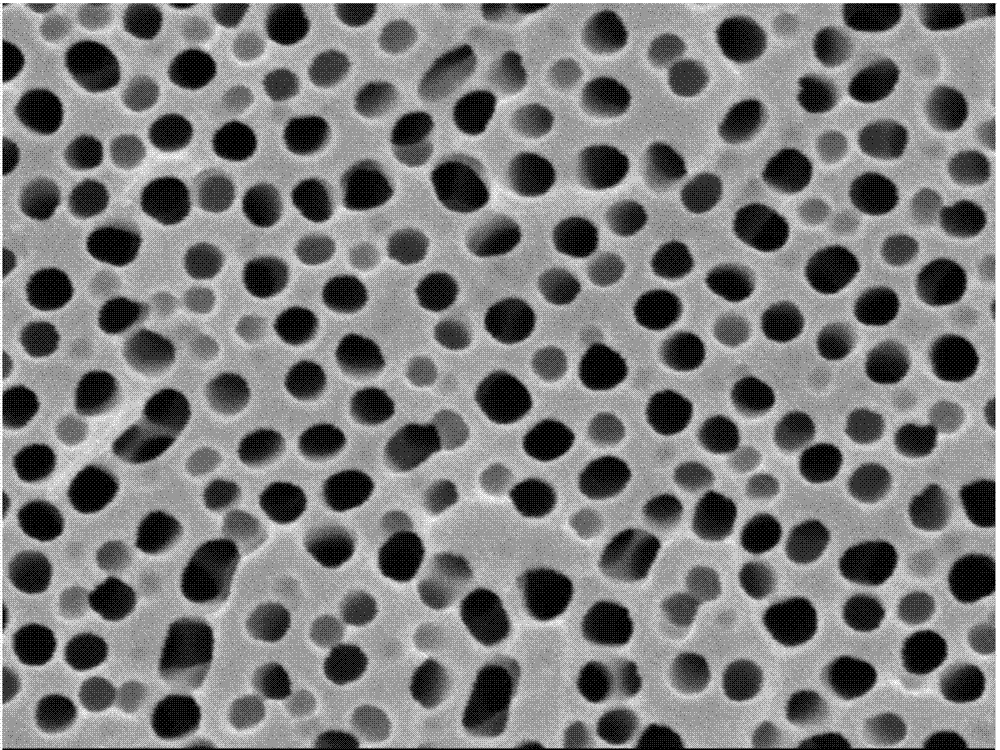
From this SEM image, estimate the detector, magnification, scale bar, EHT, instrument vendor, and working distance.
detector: SEI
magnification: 100 K X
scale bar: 100 nm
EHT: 5 kV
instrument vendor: JEOL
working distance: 7.4 mm